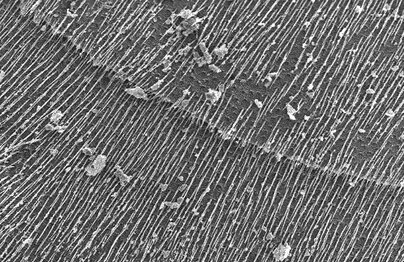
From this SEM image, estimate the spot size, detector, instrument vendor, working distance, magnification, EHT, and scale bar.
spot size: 3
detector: SE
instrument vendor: FEI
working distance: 21.4 mm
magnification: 0.101 K X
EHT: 30 kV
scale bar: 200000 nm